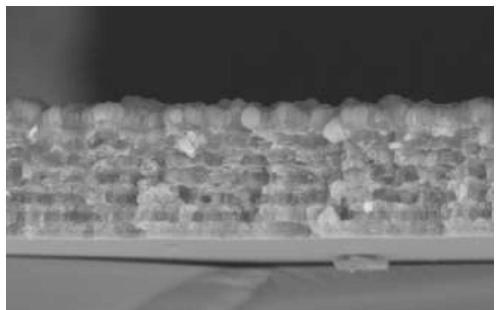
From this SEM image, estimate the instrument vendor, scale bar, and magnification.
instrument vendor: FEI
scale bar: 2000 nm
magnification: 20 K X